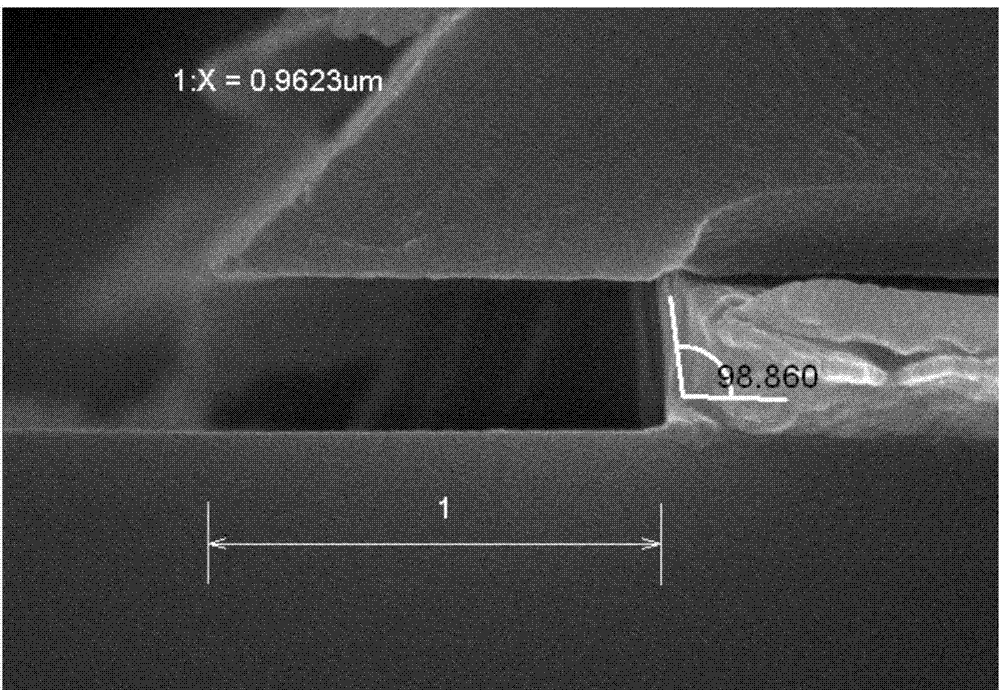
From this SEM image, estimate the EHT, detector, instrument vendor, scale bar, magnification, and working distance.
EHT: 15 kV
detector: SE(M)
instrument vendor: Hitachi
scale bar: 500 nm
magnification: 60 K X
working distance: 10.4 mm